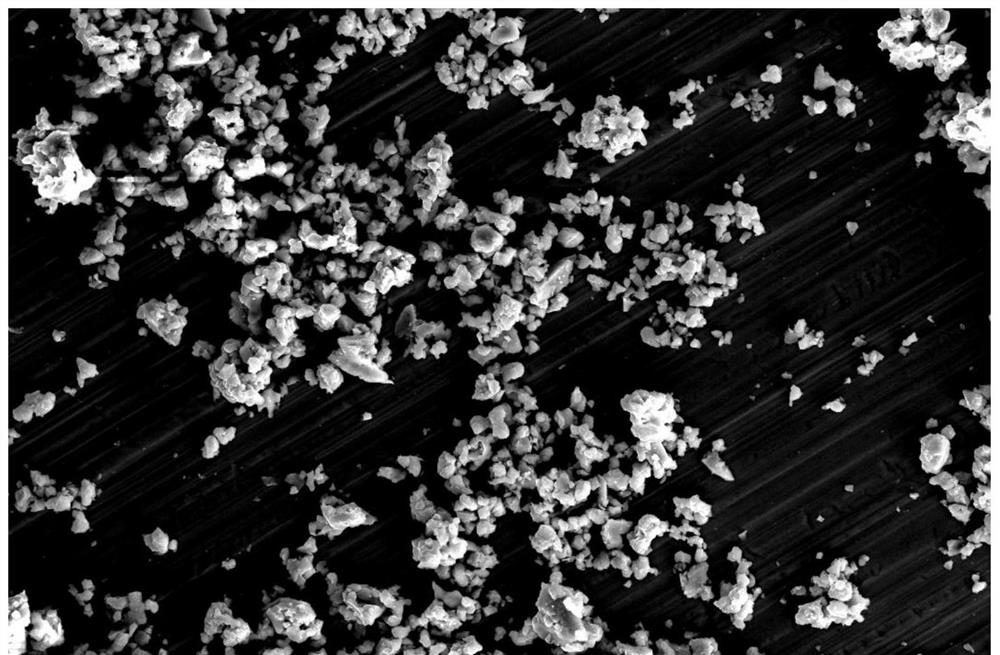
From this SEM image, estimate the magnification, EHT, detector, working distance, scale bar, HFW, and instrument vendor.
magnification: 2 K X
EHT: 30 kV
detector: ETD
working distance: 8 mm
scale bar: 50000 nm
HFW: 207 µm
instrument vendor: FEI